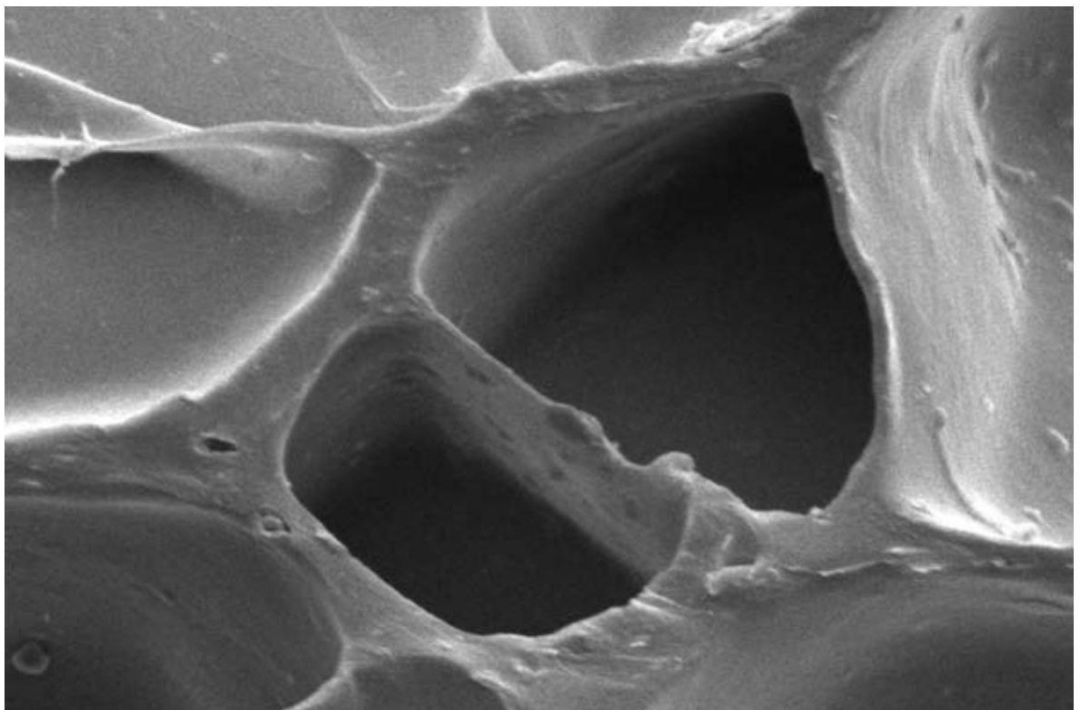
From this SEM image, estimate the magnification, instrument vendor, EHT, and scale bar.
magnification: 1 K X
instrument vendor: JEOL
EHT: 10 kV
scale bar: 10000 nm